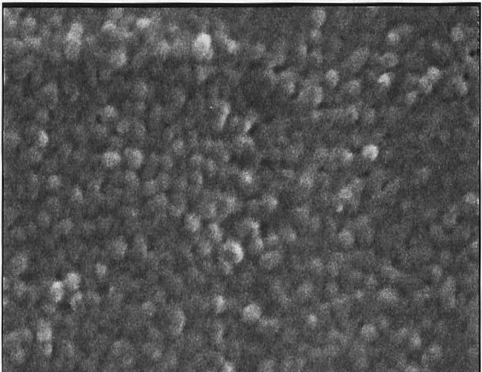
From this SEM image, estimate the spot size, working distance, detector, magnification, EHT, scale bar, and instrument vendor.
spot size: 3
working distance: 9.8 mm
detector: SE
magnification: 30 K X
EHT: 25 kV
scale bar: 500 nm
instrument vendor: FEI